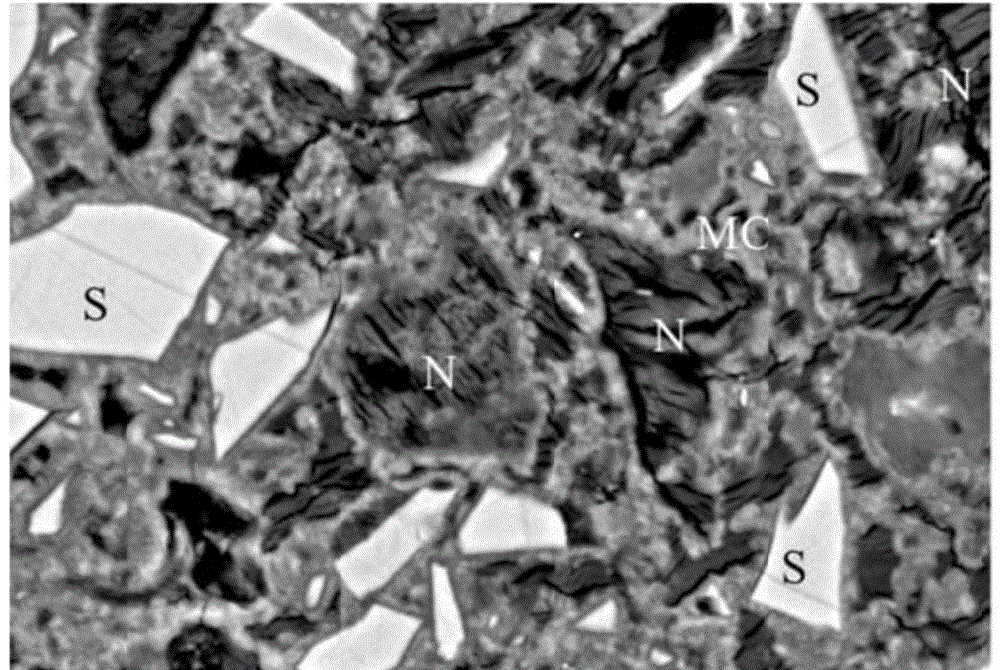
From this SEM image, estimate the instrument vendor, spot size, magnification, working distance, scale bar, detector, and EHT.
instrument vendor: JEOL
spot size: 50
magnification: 2 K X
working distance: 8 mm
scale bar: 10000 nm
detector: BEC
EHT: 15 kV